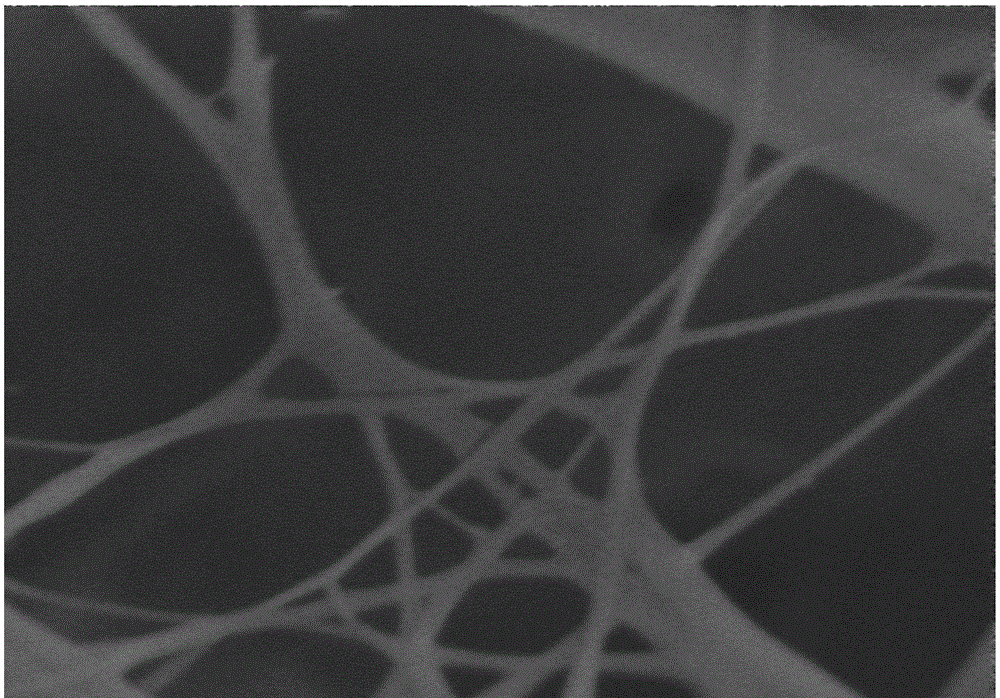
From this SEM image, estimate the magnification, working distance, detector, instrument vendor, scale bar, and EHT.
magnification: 50 K X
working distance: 6 mm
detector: HE-SE2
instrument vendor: Zeiss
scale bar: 300 nm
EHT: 5 kV